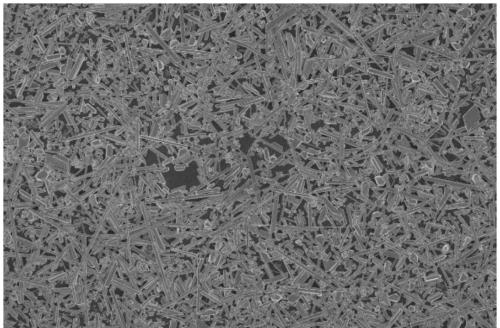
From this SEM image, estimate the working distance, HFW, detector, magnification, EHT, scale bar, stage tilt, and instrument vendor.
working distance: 4.1 mm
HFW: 104 µm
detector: TLD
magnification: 4 K X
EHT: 2 kV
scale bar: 30000 nm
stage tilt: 0°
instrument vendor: FEI